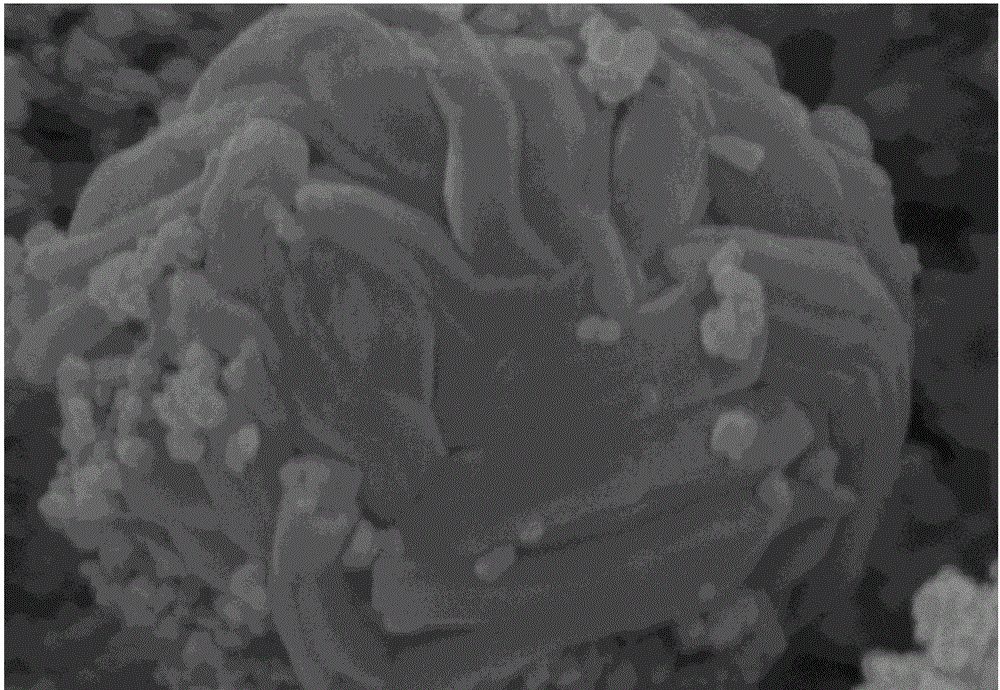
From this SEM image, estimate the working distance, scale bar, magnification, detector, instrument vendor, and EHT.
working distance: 9.1 mm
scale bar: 500 nm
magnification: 100 K X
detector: SE(M)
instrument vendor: Hitachi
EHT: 3 kV